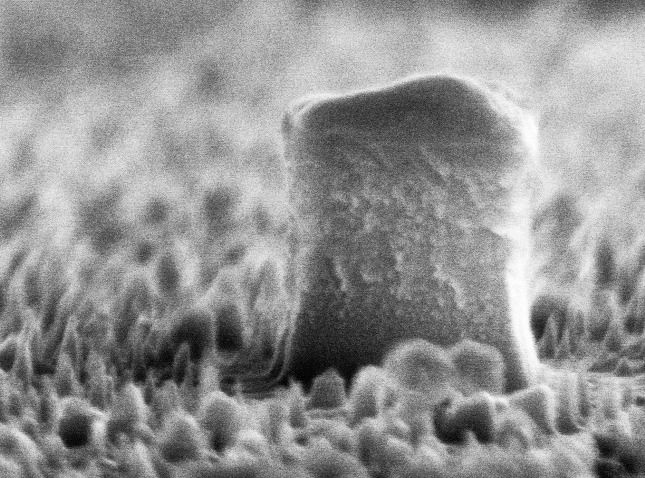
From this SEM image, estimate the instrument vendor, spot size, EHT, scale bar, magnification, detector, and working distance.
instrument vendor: FEI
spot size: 2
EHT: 10 kV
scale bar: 200 nm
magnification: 96.034 K X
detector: TLD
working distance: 5.6 mm